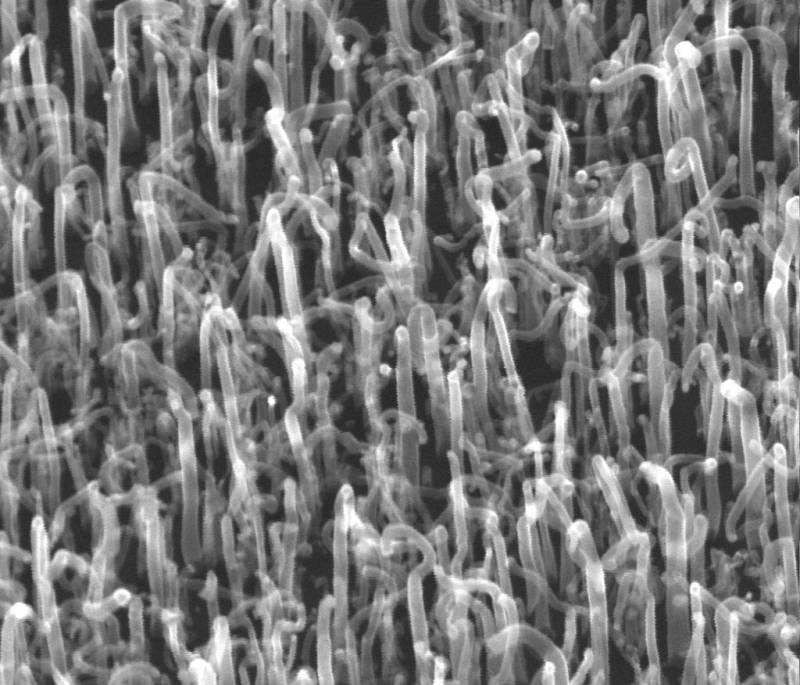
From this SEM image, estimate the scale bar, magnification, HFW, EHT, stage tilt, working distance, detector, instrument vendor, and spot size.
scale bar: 300 nm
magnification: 100 K X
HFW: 2.7 µm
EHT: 20 kV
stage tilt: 33.2°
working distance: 8.2 mm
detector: ETD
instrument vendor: FEI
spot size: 2